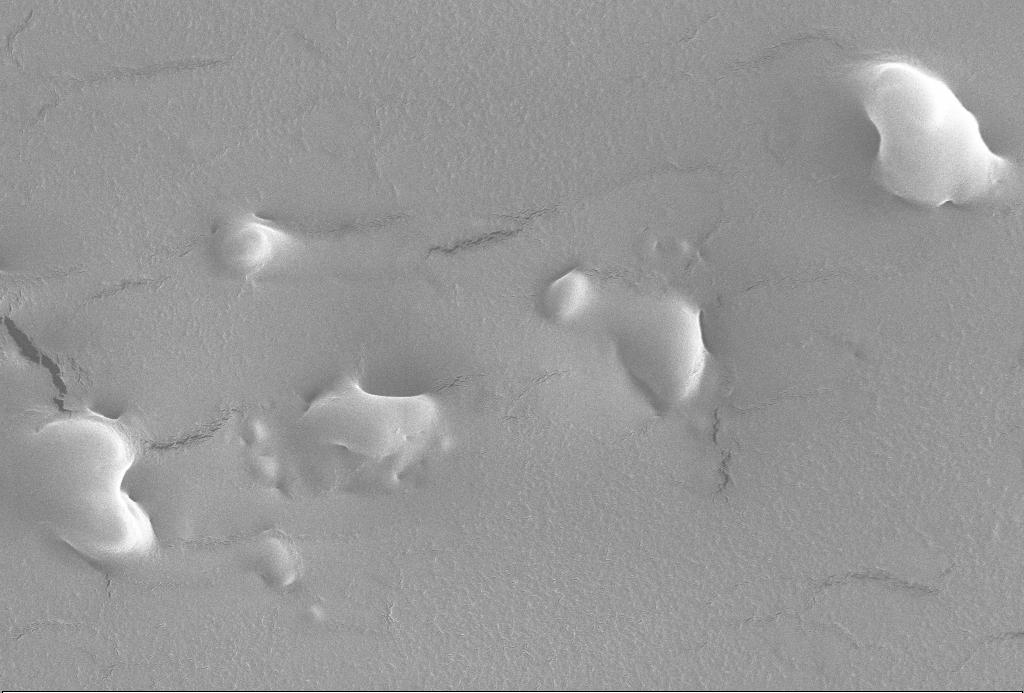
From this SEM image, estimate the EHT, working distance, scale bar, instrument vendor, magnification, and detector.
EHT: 20 kV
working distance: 8 mm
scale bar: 1000 nm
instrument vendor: Zeiss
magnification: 10 K X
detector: SE1